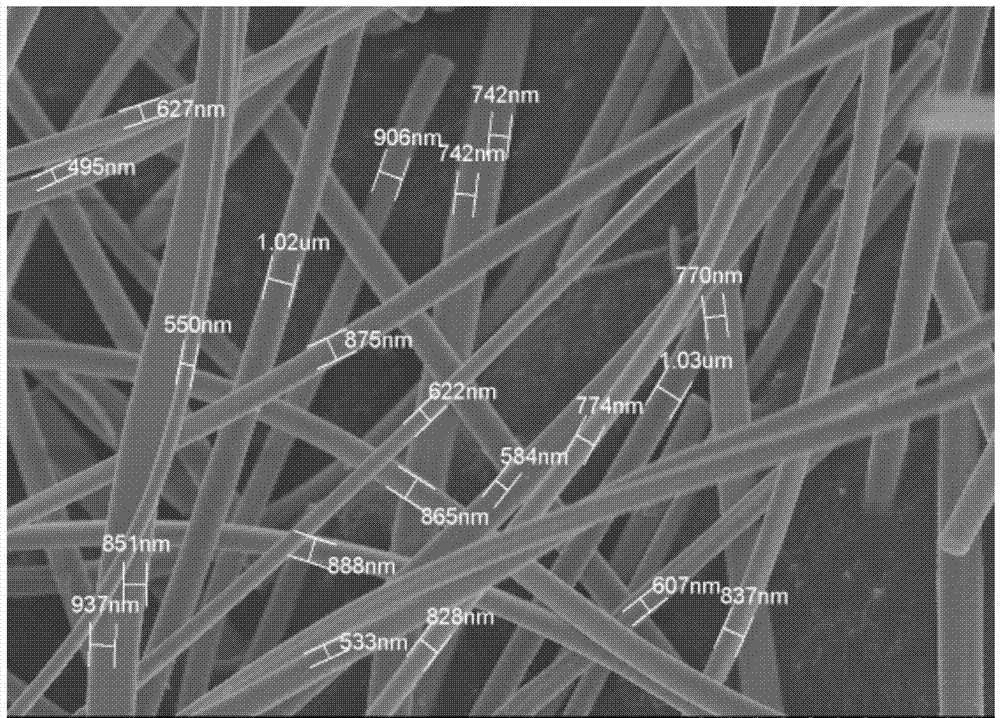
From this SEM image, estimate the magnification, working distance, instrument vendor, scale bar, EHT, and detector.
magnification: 3.5 K X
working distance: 10 mm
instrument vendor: Hitachi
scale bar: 10000 nm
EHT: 7 kV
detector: SE(M)